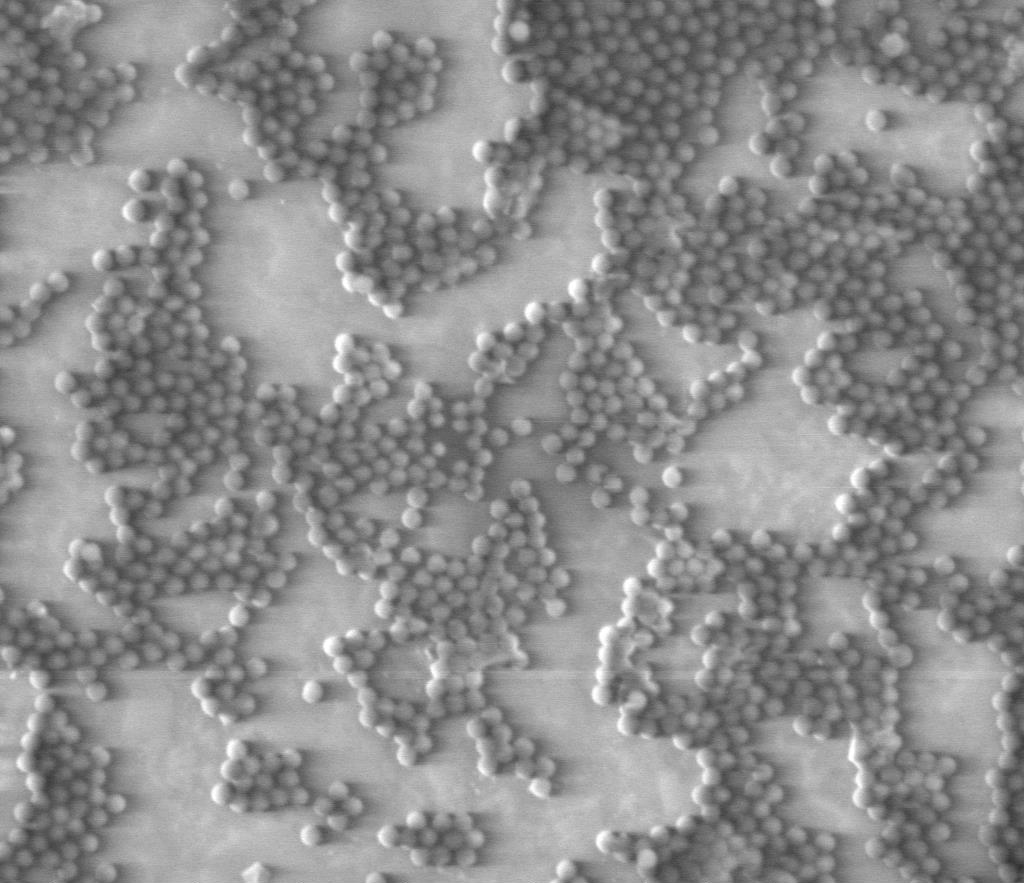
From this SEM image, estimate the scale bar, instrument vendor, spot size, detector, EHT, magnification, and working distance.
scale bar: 4000 nm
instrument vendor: FEI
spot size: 4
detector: LVD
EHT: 4 kV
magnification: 16 K X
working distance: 5.7 mm